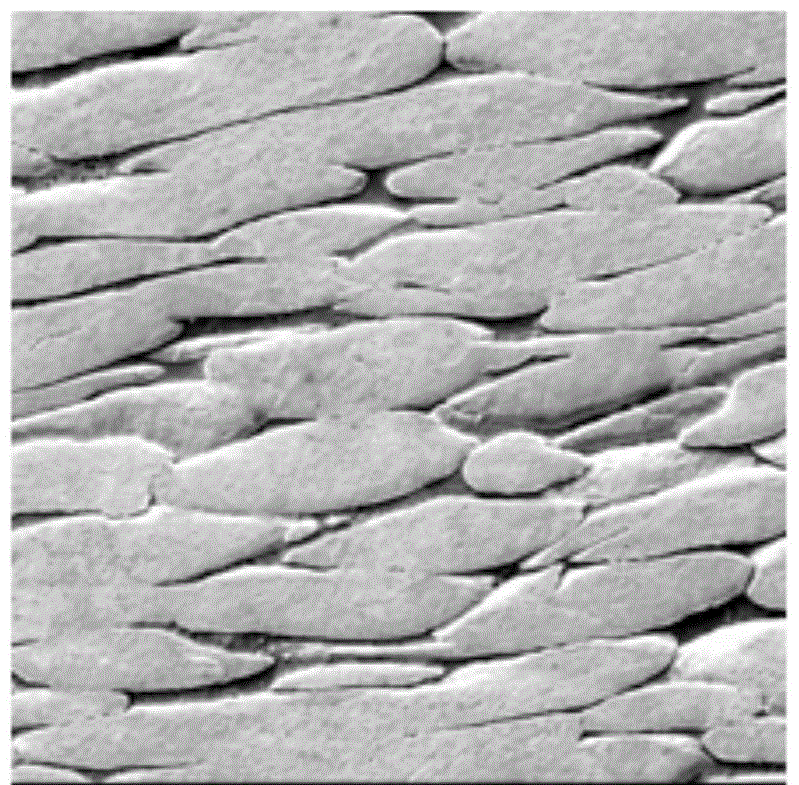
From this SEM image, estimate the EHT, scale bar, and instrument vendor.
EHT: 20 kV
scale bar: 60000 nm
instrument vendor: JEOL